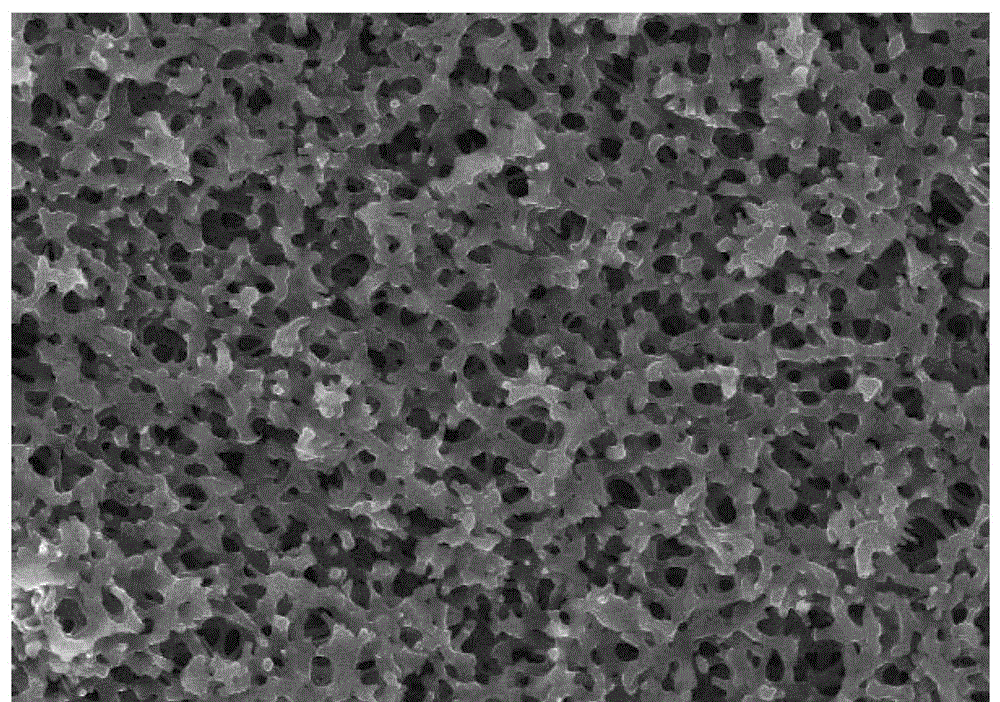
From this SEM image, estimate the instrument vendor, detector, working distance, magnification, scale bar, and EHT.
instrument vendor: Hitachi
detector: SE(M)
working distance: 7.7 mm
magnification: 5 K X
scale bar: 10000 nm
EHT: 10 kV